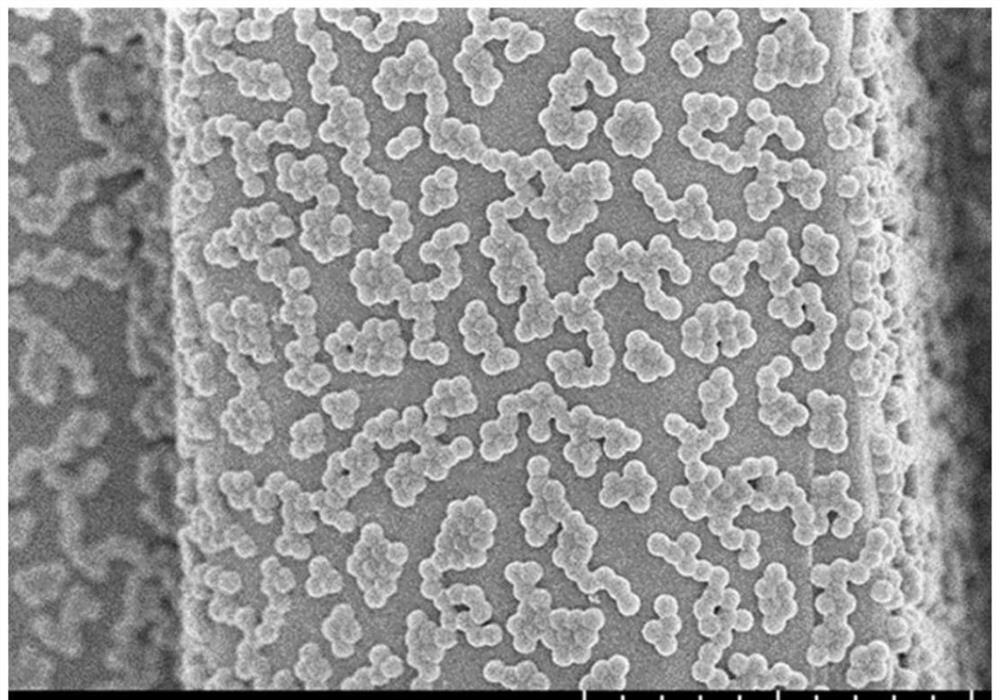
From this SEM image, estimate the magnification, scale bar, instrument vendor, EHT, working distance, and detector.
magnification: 10 K X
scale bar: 5000 nm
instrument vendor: Hitachi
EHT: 3 kV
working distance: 6.4 mm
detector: SE(UL)